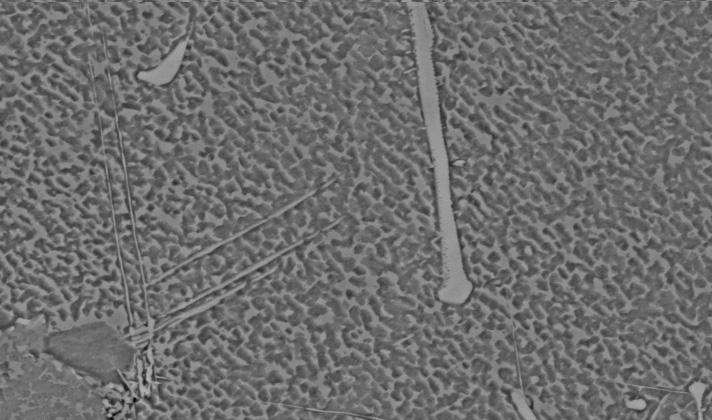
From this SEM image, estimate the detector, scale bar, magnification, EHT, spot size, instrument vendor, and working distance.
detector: BSE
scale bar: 10000 nm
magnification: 2 K X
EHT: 20 kV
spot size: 4.1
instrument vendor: FEI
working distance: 10.8 mm